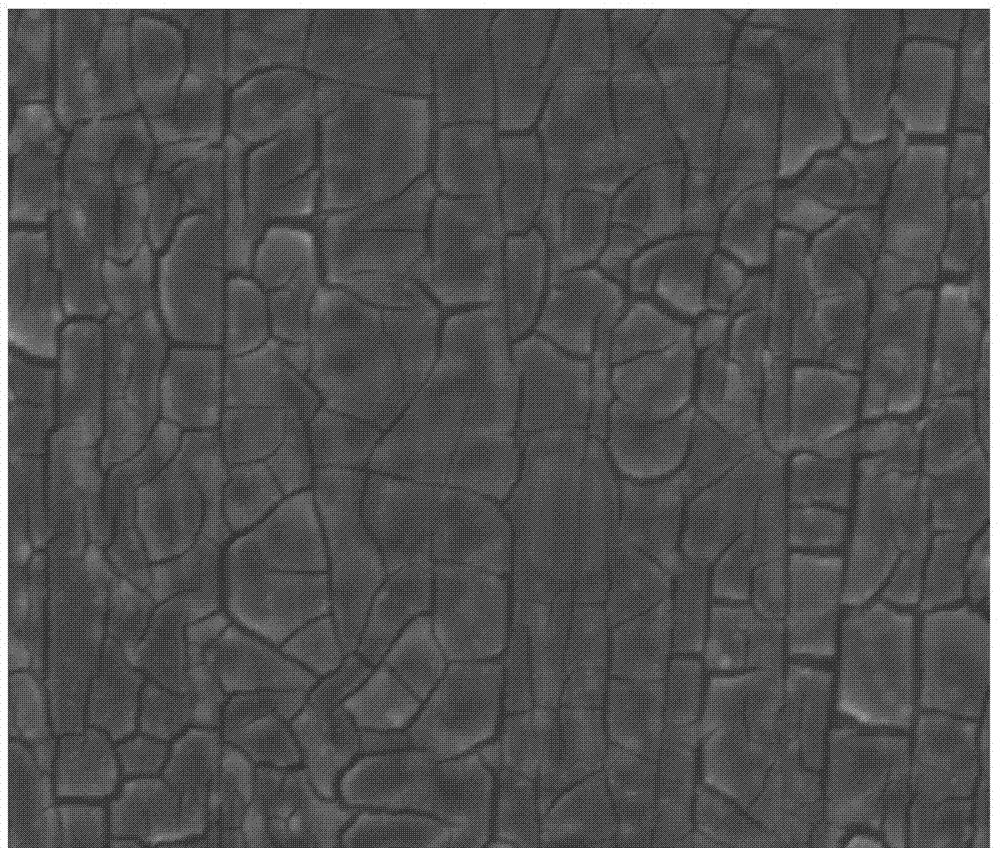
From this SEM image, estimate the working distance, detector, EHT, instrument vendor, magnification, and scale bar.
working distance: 11.4 mm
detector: SE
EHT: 20 kV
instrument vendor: FEI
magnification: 3 K X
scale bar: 30000 nm